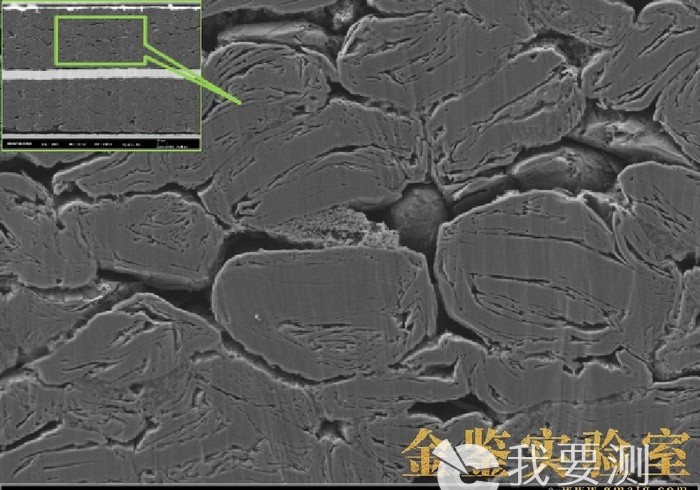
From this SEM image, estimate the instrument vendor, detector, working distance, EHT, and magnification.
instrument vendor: Zeiss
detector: SE2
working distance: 5.1 mm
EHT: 5 kV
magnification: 2 K X